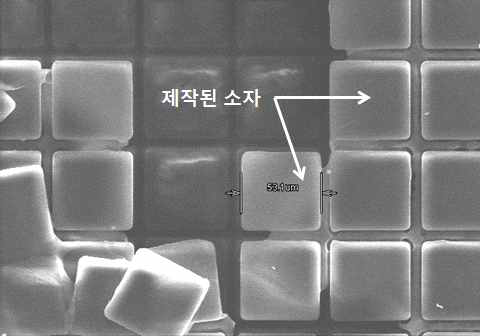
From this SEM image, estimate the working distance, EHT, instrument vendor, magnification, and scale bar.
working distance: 10.5 mm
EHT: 25 kV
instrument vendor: Hitachi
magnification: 0.4 K X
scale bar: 100000 nm